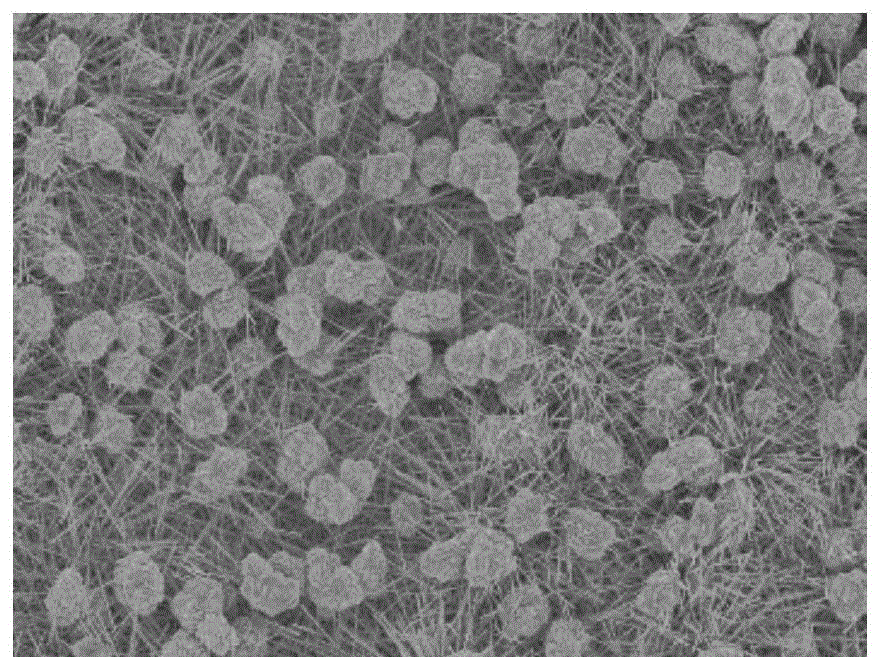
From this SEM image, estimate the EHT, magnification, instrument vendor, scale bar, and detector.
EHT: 8 kV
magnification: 1 K X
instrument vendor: Hitachi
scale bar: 50000 nm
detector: SE(U)